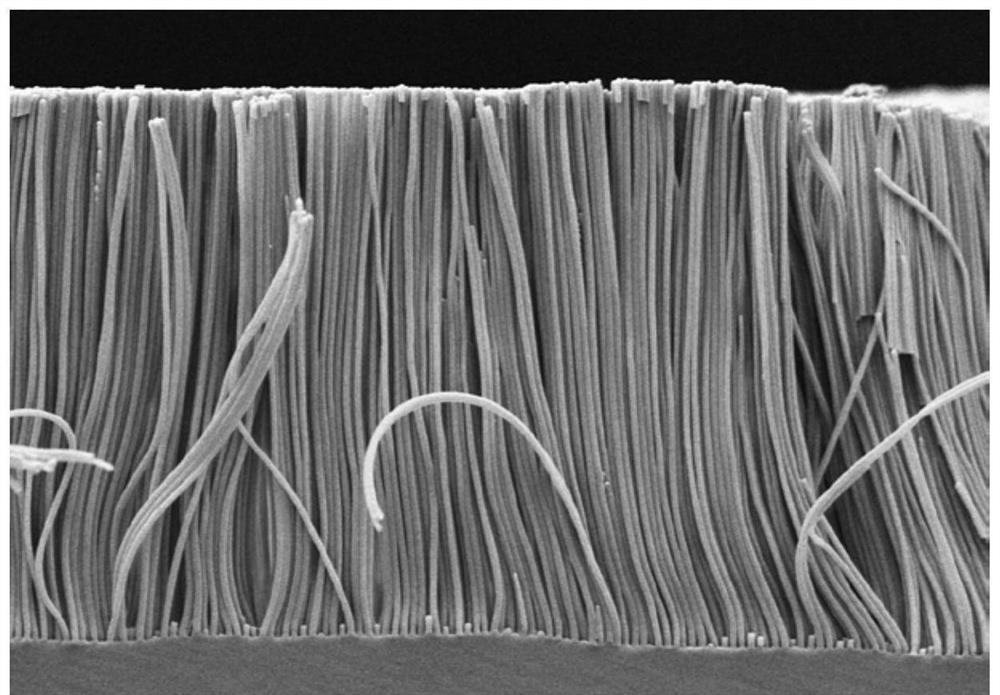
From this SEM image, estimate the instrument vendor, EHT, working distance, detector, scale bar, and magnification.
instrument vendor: Hitachi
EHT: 5 kV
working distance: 9.1 mm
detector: SE(M,LA0)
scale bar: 20000 nm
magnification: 2.5 K X